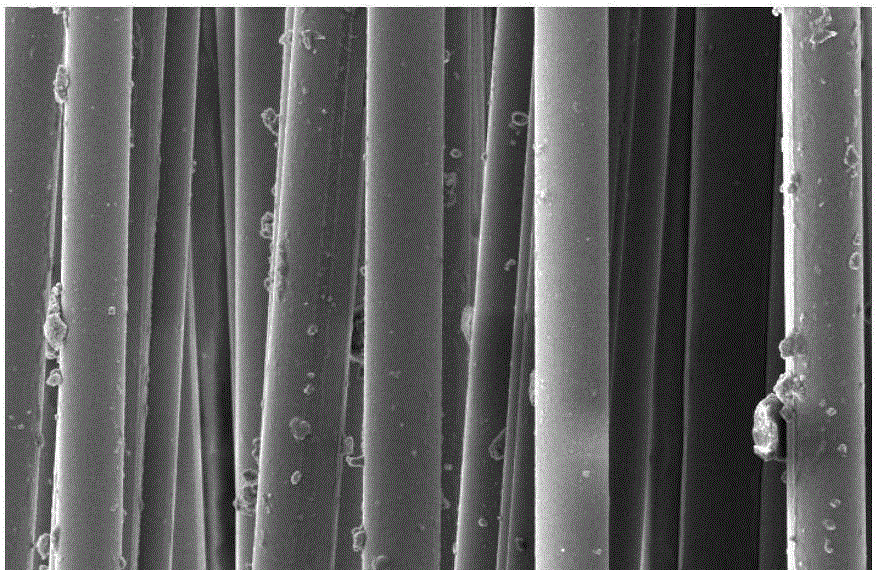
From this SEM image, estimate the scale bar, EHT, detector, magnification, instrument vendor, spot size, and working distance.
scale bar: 50000 nm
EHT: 10 kV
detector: SE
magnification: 0.5 K X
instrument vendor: FEI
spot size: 3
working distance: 6.6 mm